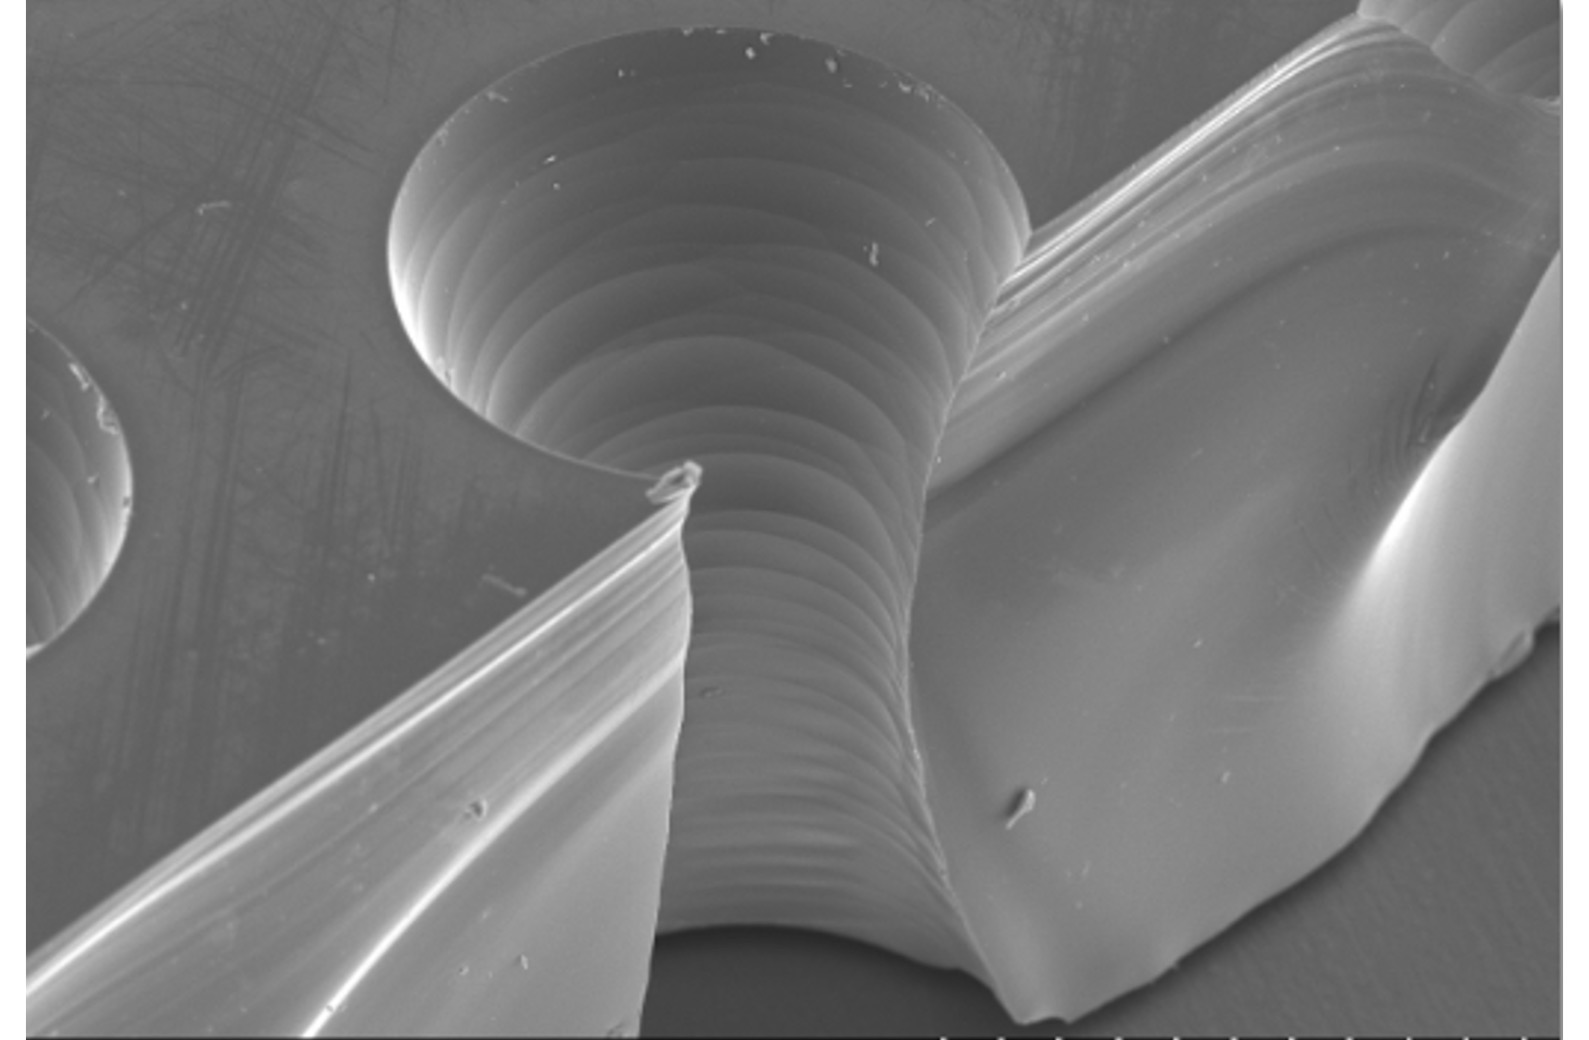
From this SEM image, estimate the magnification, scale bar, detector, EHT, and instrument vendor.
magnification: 0.16 K X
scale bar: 300000 nm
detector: SE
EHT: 15 kV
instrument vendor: Hitachi